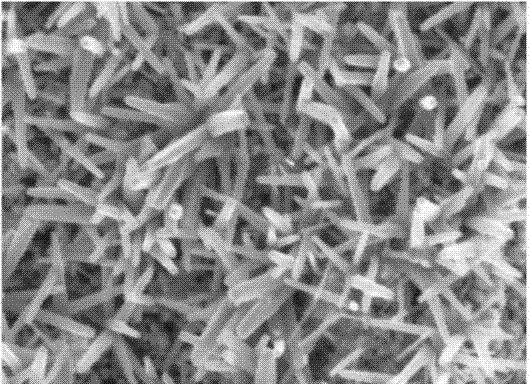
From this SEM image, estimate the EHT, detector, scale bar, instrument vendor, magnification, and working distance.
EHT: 5 kV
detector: InLens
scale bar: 200 nm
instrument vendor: Zeiss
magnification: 30 K X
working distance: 5 mm